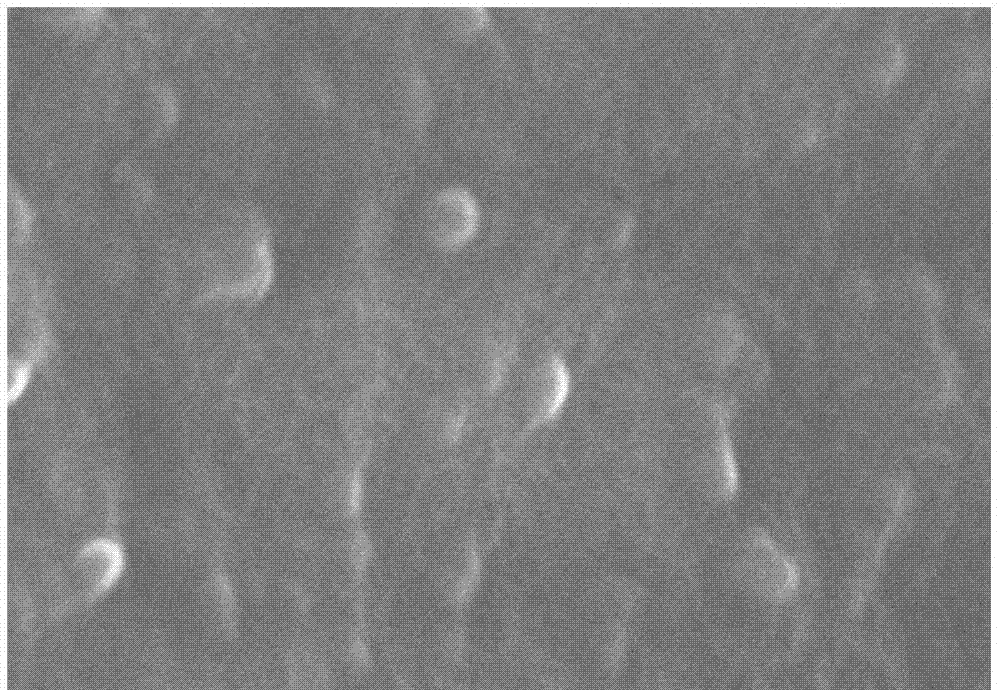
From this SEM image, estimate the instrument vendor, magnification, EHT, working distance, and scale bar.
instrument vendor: Hitachi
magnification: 60 K X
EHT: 20 kV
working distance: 12.3 mm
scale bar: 500 nm